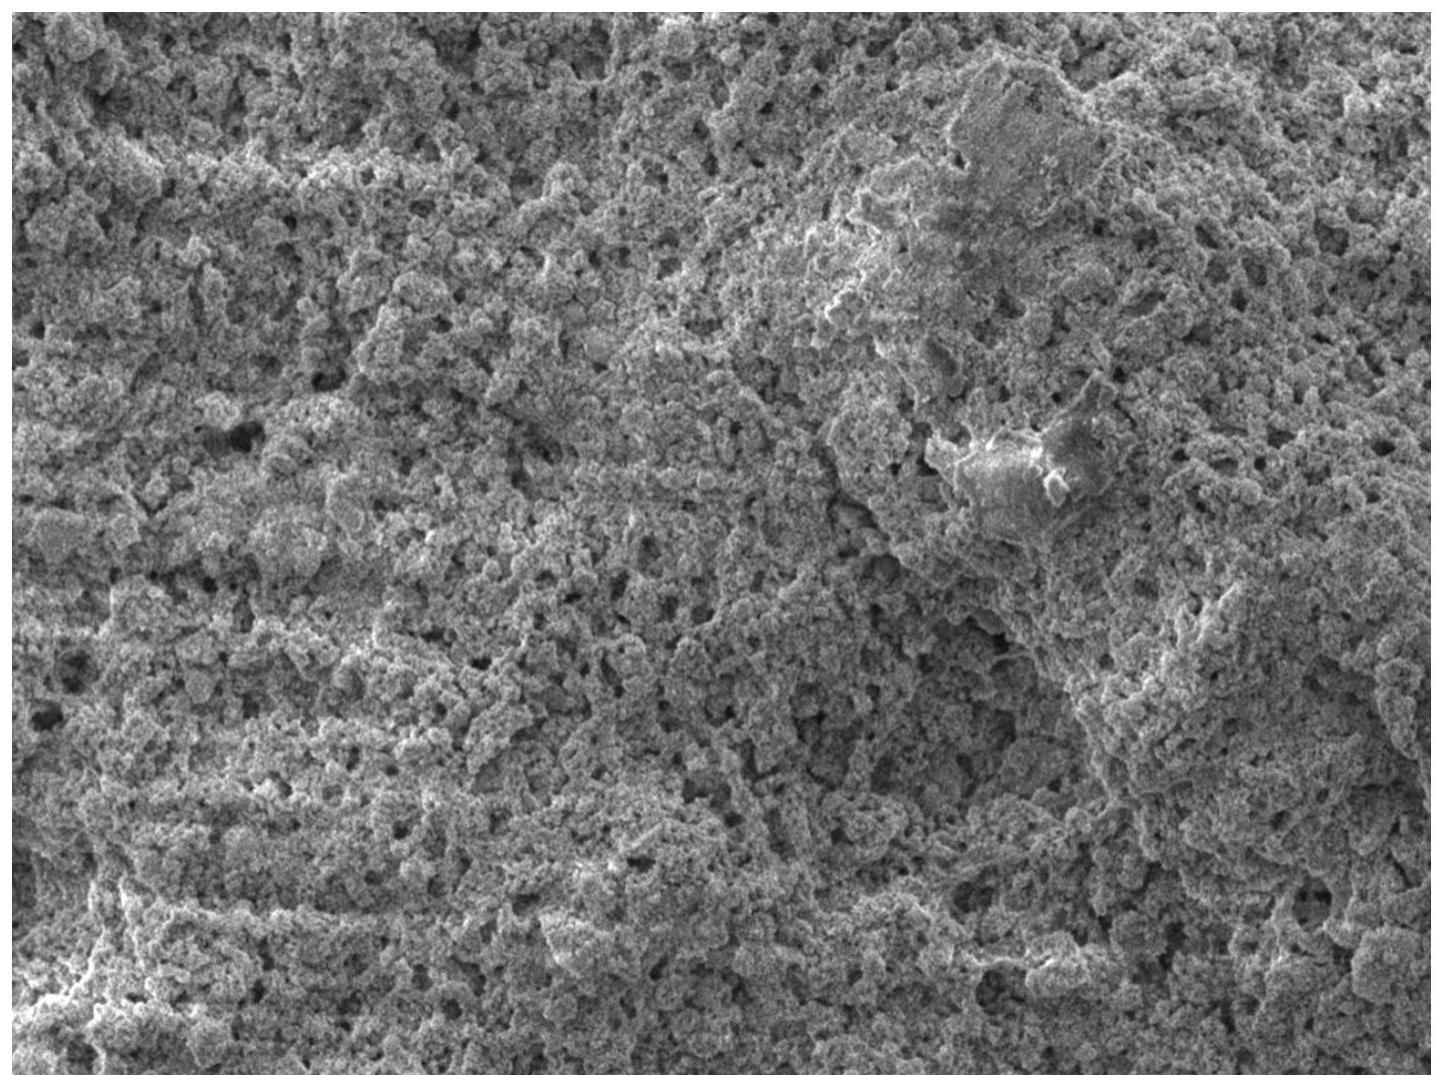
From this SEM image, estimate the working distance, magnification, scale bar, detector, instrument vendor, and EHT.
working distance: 10.5 mm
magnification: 5 K X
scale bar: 1000 nm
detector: LED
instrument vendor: JEOL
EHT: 2 kV